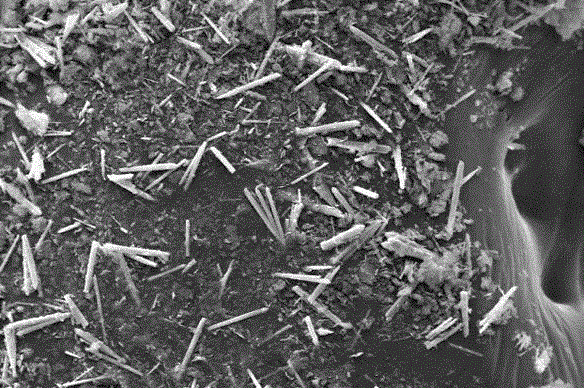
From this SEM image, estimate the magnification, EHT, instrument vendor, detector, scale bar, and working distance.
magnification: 2 K X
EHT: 20 kV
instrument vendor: Zeiss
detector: SE1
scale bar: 10000 nm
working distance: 8.5 mm